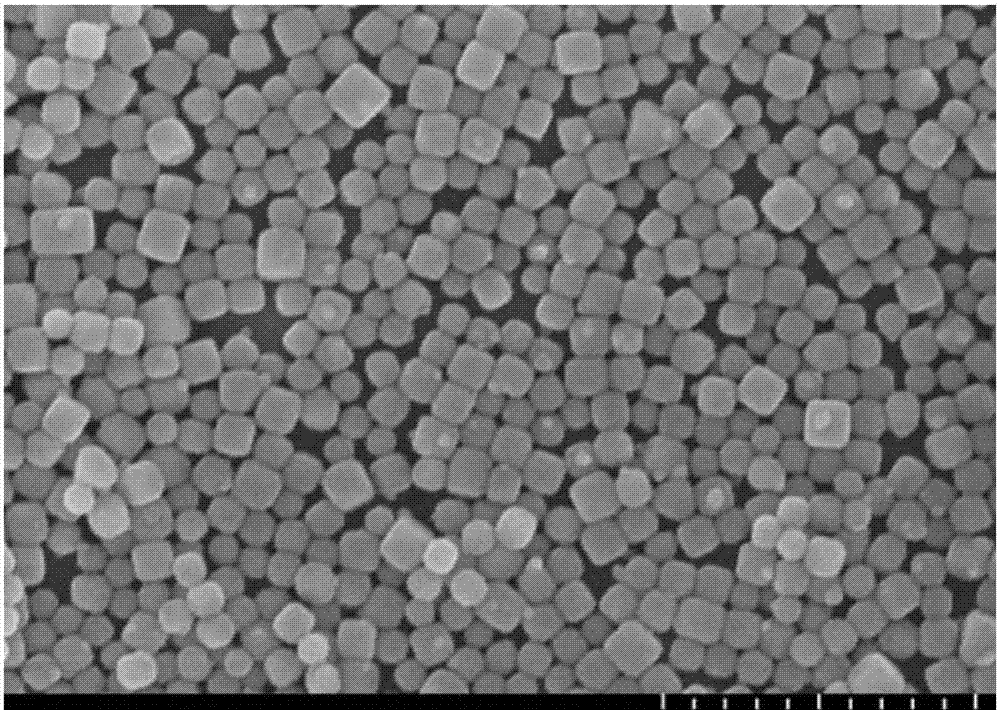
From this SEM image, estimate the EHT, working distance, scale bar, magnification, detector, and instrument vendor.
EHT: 10 kV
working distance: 9.5 mm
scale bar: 1000 nm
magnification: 40 K X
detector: SE(U)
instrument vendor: Hitachi